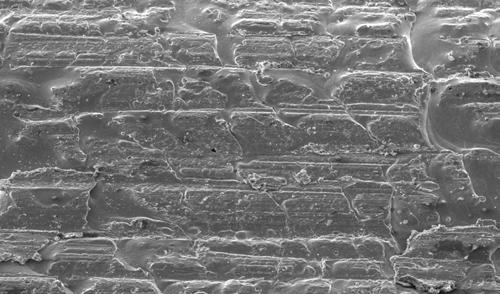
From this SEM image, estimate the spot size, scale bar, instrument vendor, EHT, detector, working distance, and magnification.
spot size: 4.4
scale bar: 200000 nm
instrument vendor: FEI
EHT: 25 kV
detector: SE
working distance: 30.8 mm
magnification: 0.15 K X